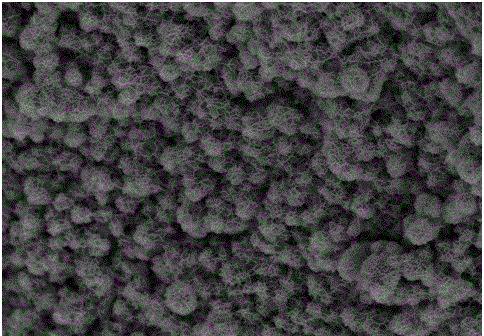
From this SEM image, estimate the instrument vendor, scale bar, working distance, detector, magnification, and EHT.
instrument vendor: Hitachi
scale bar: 5000 nm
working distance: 9 mm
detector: SE(M)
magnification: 10 K X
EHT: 5 kV